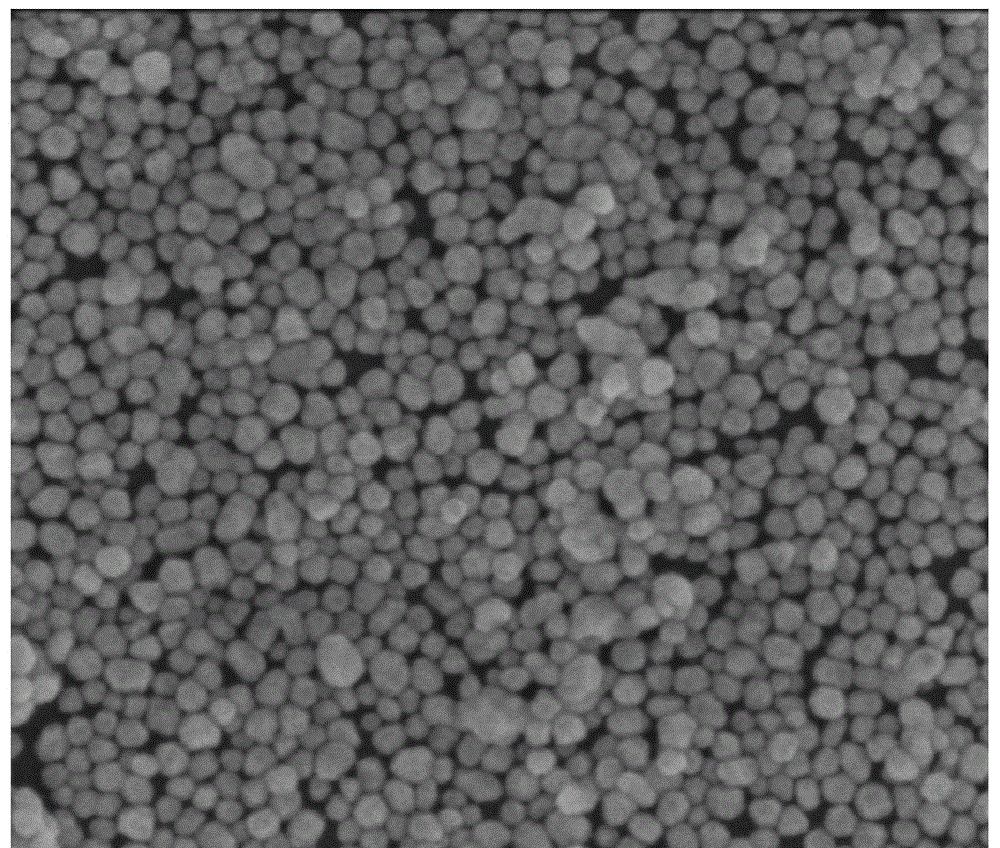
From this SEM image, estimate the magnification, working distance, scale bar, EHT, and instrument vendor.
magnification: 100 K X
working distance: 4.8 mm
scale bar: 500 nm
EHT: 5 kV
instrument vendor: FEI